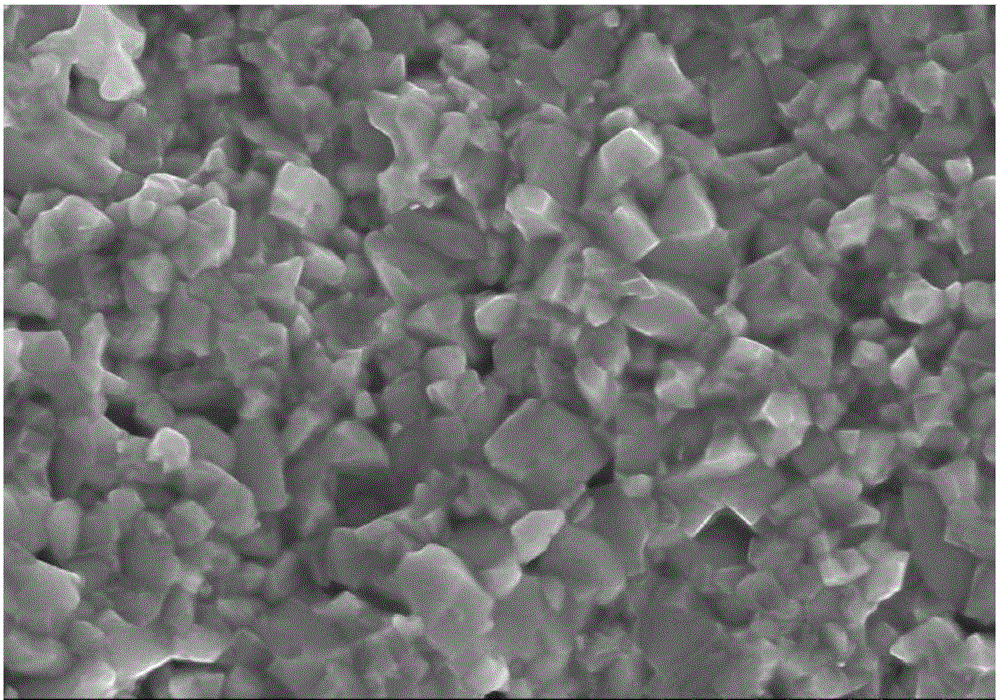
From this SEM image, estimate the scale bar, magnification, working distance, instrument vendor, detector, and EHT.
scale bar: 2000 nm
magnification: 20 K X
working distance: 11.8 mm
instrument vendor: Hitachi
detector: SE(M)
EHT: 15 kV